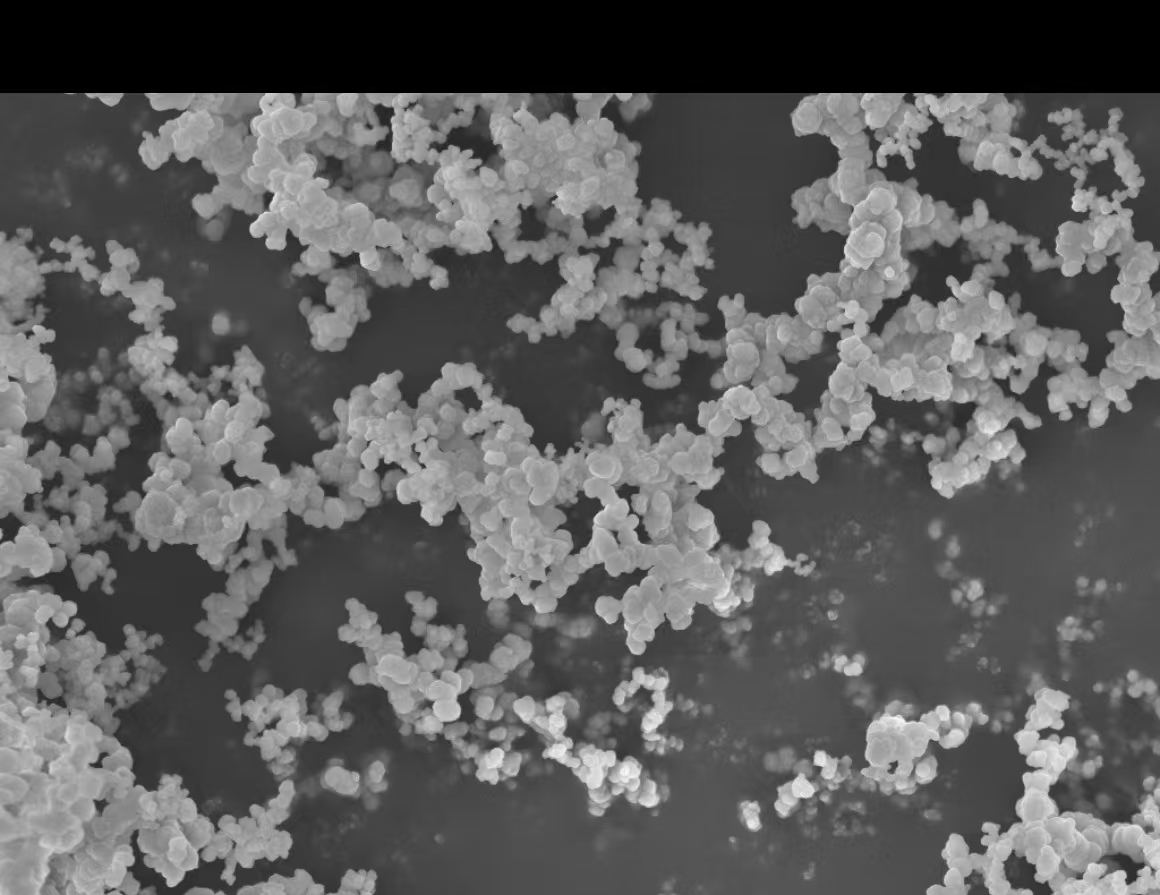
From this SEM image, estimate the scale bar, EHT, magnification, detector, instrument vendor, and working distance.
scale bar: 1000 nm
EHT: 5 kV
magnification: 10 K X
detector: InLens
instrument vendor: Zeiss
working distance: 4.1 mm